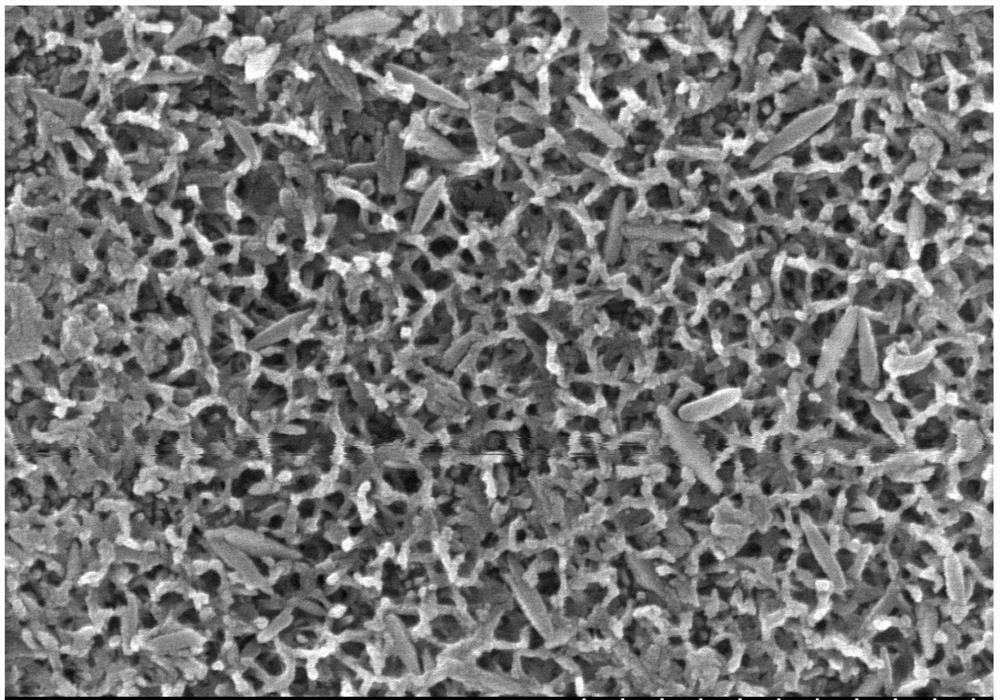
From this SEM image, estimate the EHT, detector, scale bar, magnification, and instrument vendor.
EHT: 8 kV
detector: SE(M)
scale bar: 1000 nm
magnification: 50 K X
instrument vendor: Hitachi